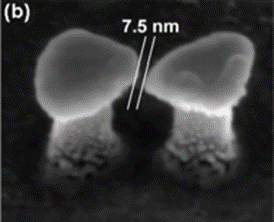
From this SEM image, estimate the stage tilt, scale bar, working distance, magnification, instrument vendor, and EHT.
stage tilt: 36°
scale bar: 100 nm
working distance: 3.1 mm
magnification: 400 K X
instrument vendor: FEI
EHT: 5 kV